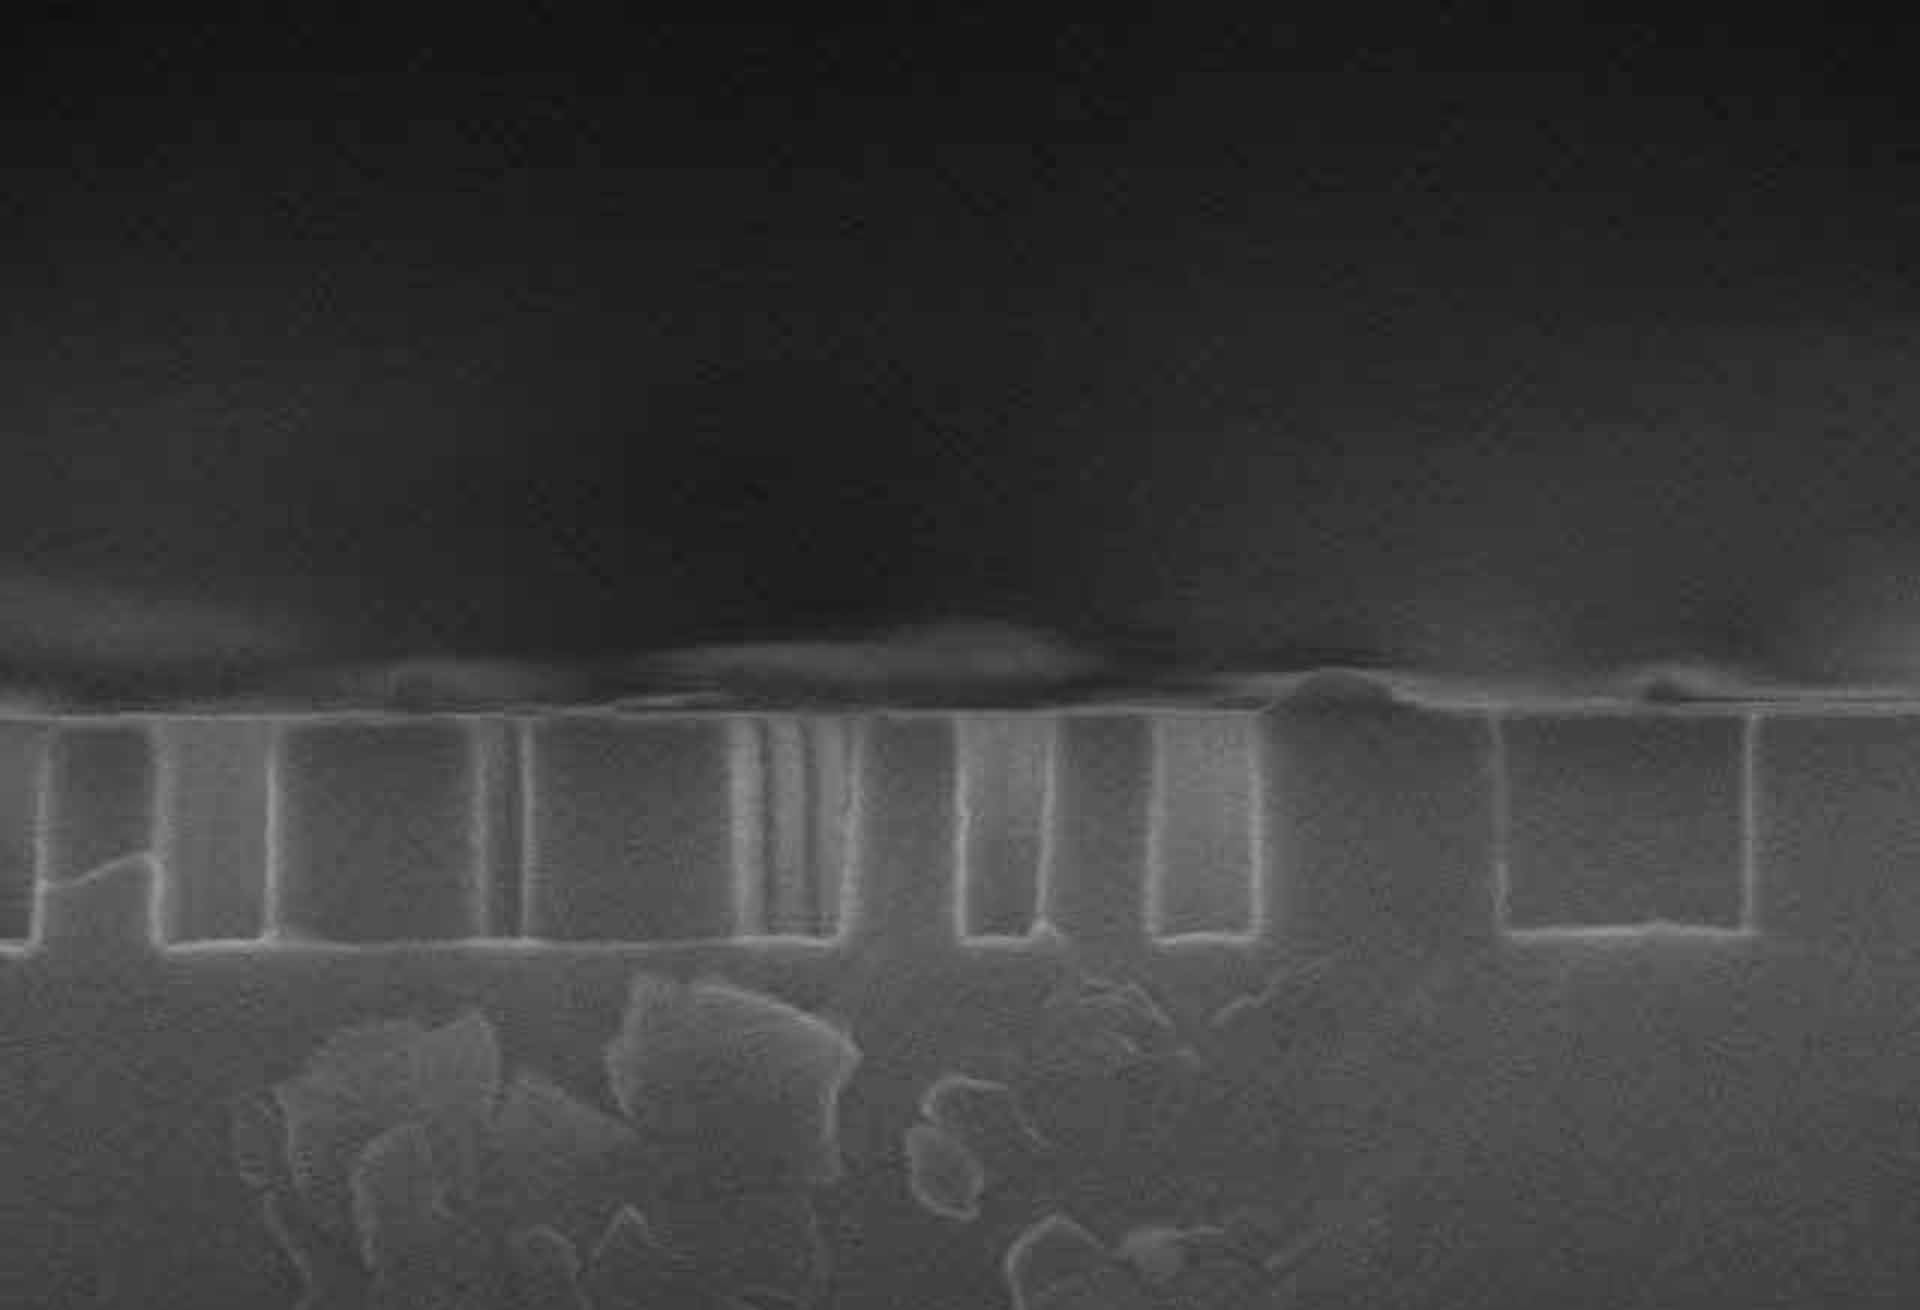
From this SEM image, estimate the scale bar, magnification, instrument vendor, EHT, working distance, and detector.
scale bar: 1000 nm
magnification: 50 K X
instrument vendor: Hitachi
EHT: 15 kV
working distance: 15.3 mm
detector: SE(M)